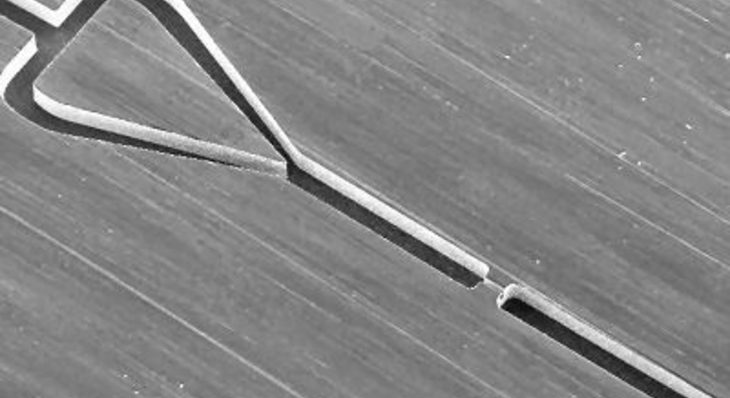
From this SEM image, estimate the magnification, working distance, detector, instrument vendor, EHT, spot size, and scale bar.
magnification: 0.1 K X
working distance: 11 mm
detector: SE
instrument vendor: FEI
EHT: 25 kV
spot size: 3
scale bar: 500000 nm